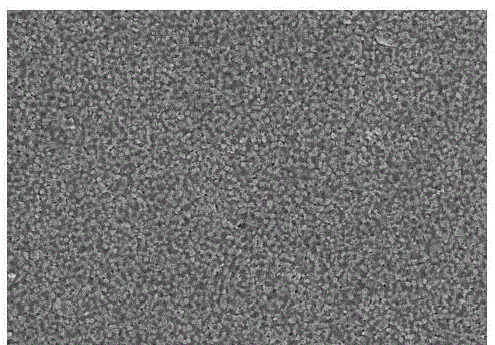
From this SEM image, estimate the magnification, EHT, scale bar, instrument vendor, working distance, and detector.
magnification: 10 K X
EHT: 15 kV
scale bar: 5000 nm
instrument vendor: Hitachi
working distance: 8.7 mm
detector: SE(M)